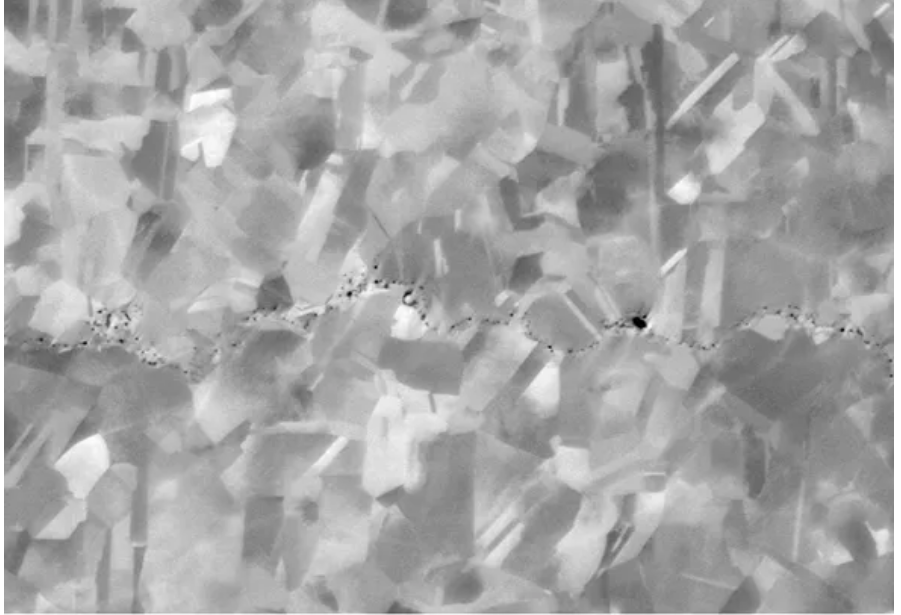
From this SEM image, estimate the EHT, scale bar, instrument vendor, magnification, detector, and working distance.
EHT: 8 kV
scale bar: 1000 nm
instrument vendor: Zeiss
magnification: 8 K X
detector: NTS BSD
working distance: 6.5 mm